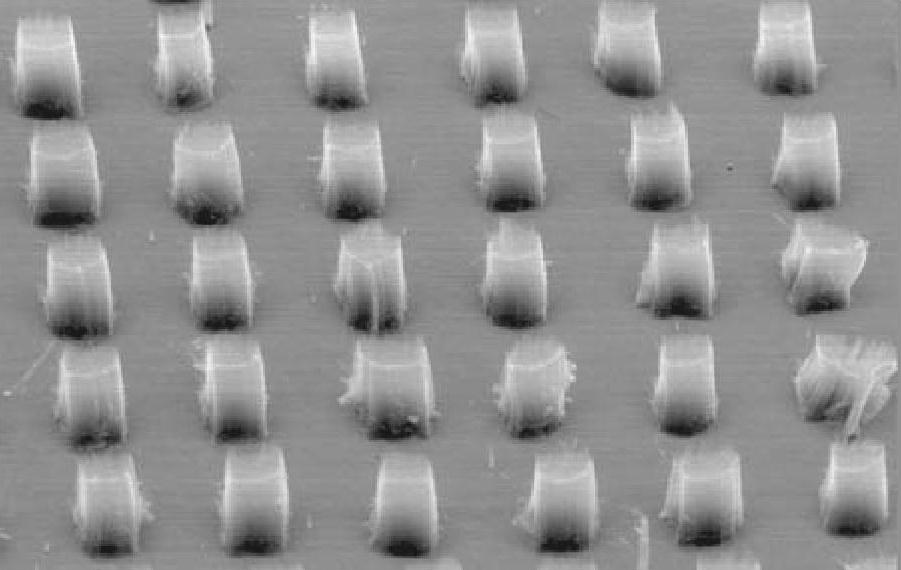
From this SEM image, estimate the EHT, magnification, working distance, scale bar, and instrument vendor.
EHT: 10 kV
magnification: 0.2 K X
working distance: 12 mm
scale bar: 100000 nm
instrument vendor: JEOL